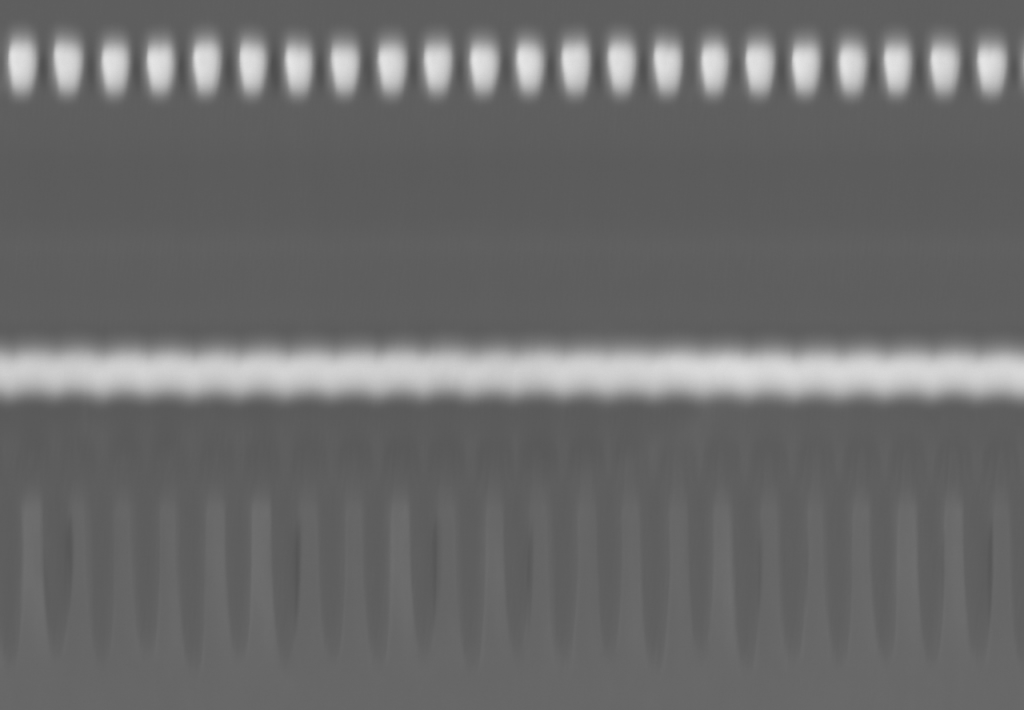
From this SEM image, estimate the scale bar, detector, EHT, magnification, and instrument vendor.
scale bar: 300 nm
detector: ZC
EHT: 200 kV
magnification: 100 K X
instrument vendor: Hitachi